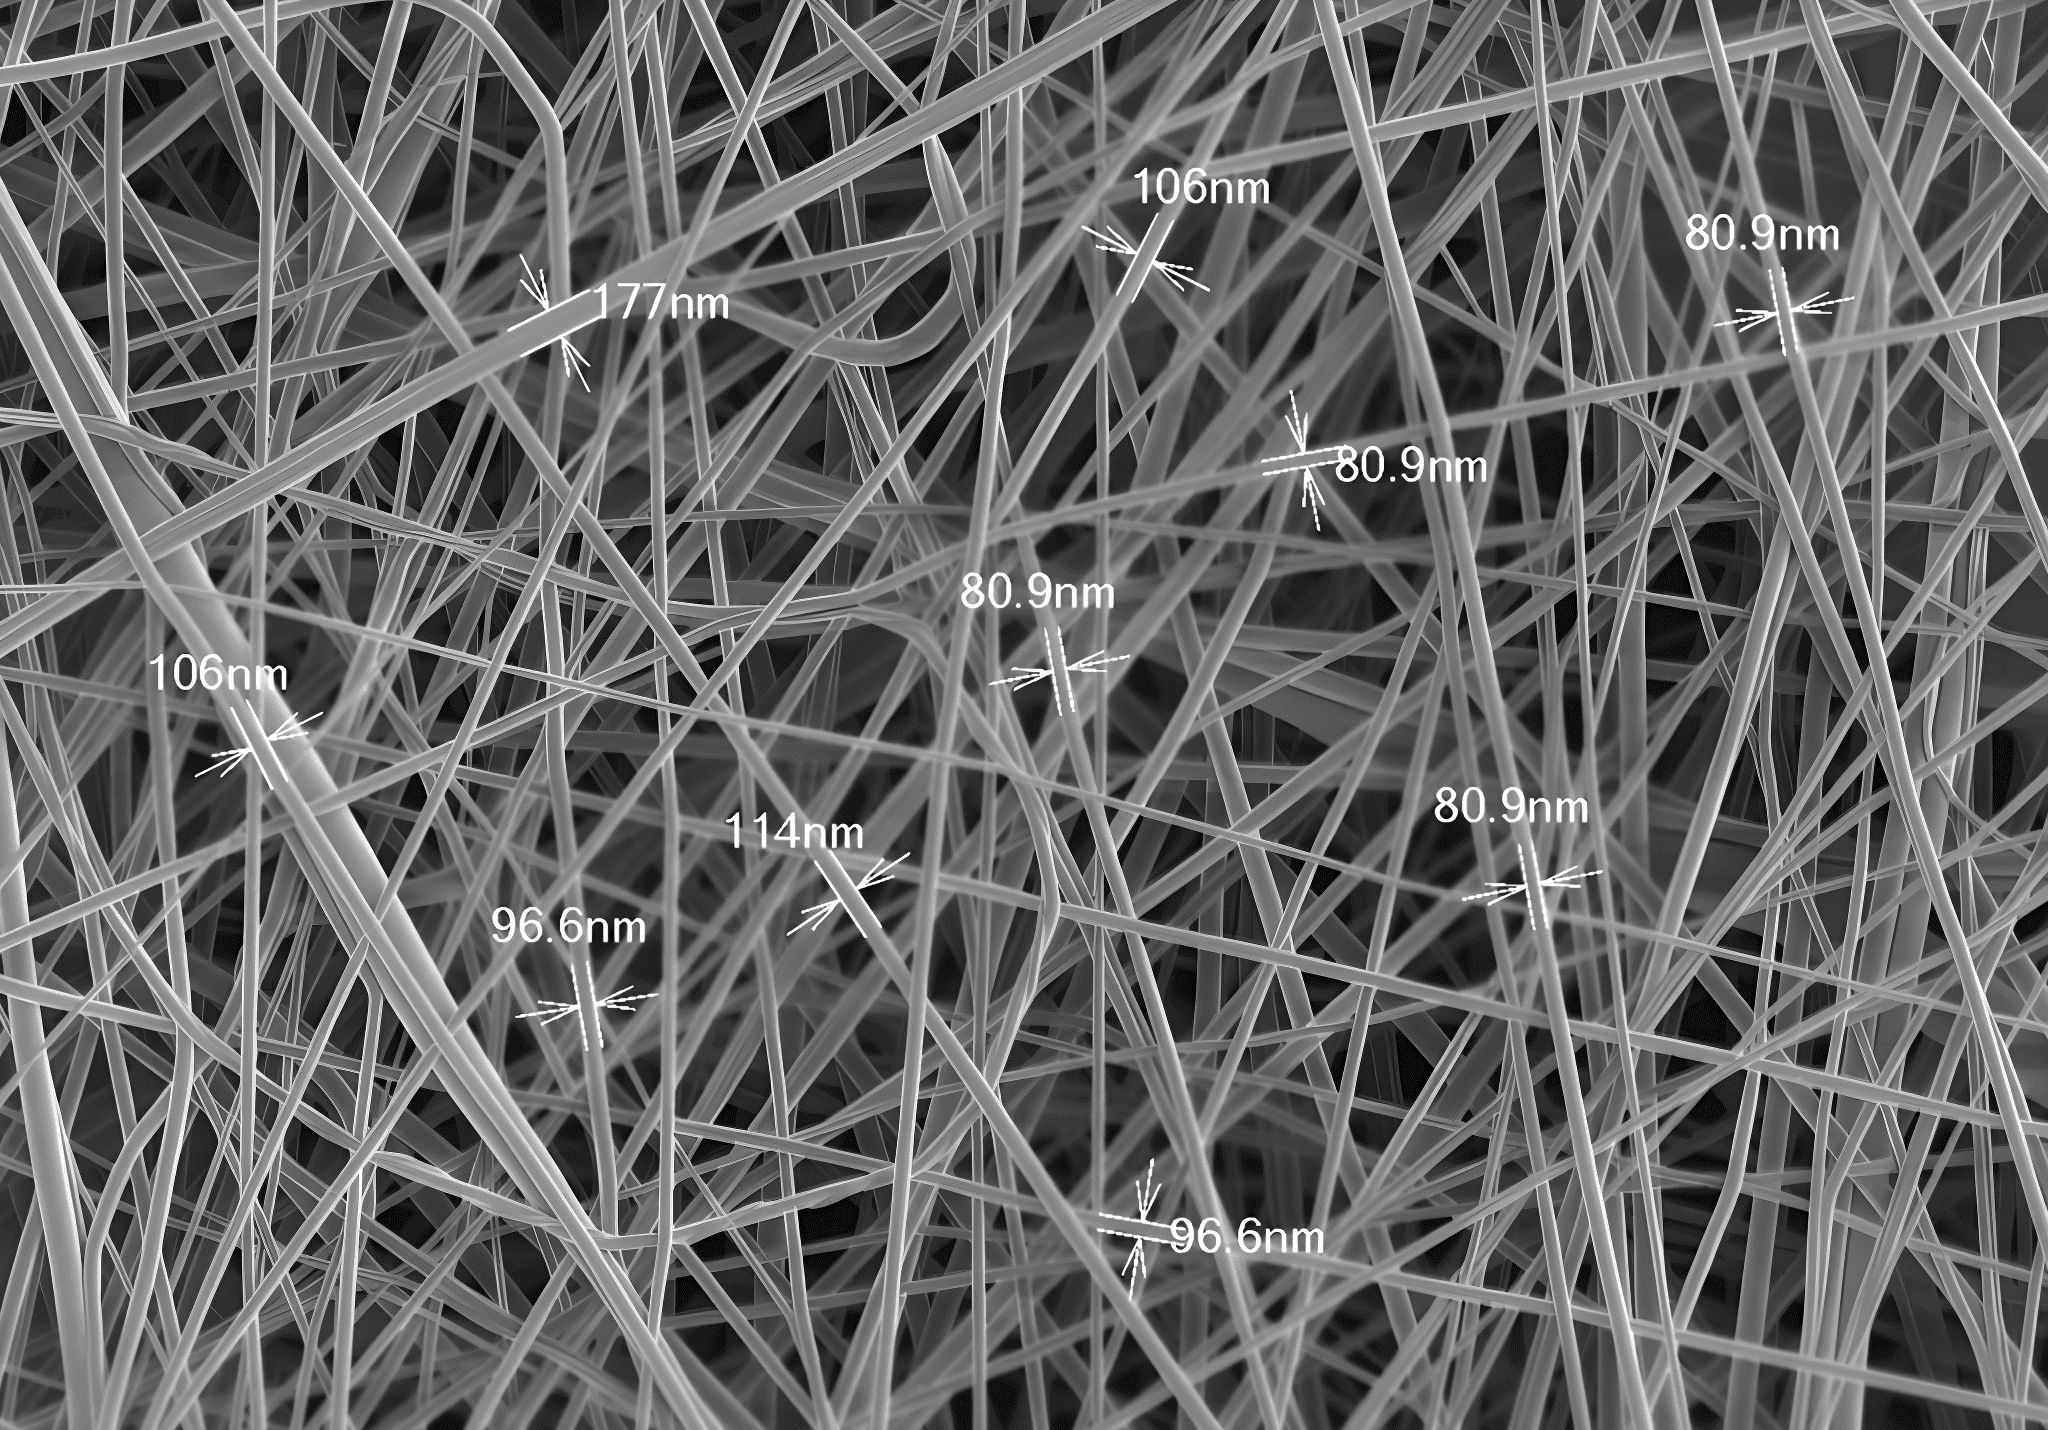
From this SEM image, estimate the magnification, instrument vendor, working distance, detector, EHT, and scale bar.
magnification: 10 K X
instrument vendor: Hitachi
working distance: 9.3 mm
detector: SE(UL)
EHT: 1 kV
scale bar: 5000 nm